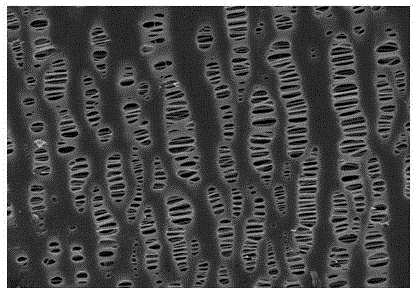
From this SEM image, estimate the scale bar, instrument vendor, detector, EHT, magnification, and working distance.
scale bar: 2000 nm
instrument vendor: Hitachi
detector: SE(M)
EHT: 2 kV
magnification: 20 K X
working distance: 4.5 mm